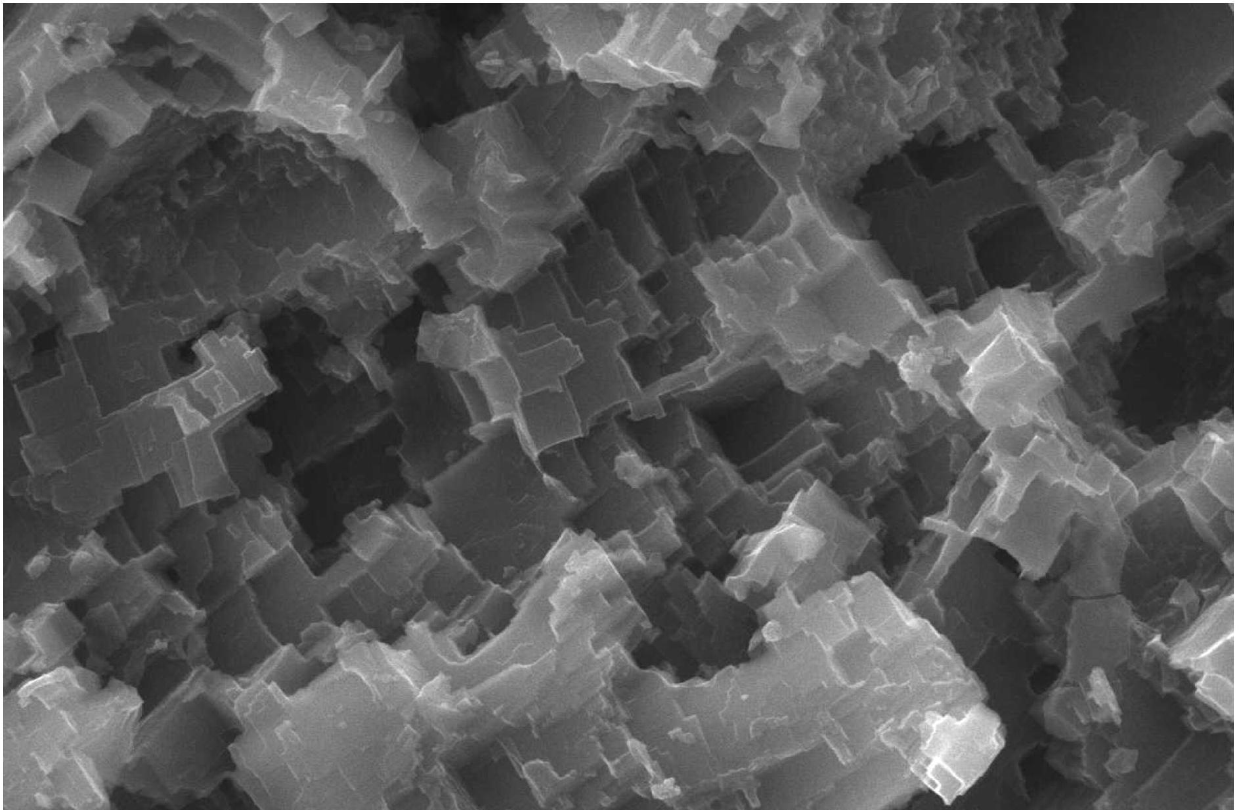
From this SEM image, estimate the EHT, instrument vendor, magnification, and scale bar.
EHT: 20 kV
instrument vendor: JEOL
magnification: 5 K X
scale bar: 5000 nm